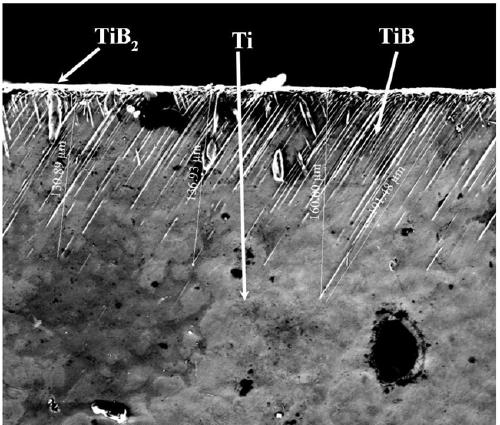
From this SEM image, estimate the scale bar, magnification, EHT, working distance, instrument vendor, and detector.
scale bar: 100000 nm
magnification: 0.8 K X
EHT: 20 kV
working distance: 10.6 mm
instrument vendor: FEI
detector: ETD